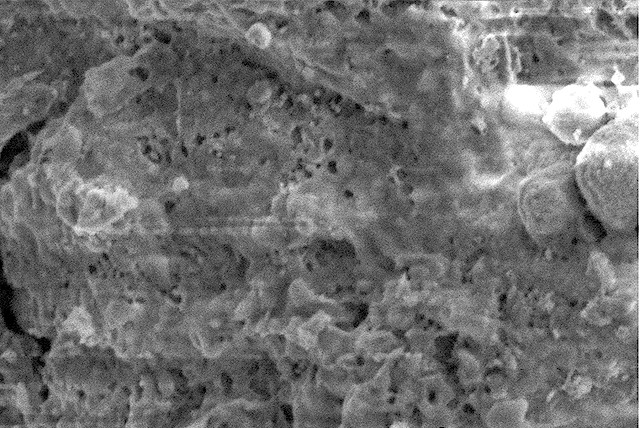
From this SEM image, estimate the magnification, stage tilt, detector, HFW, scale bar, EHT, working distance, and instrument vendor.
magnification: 35 K X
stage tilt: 0°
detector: ETD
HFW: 5.92 µm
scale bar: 1000 nm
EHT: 2 kV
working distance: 4.1 mm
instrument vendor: FEI